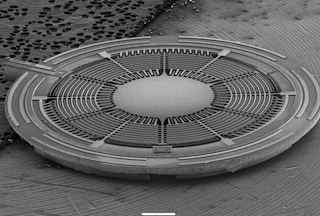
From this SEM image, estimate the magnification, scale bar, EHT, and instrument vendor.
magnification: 0.013 K X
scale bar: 1000000 nm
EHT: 10 kV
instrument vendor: JEOL